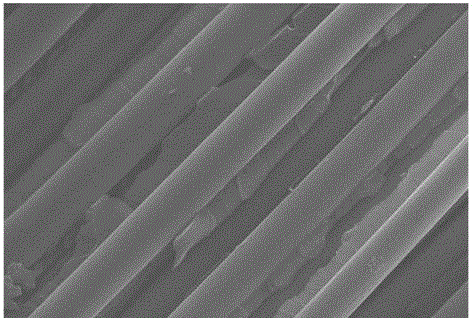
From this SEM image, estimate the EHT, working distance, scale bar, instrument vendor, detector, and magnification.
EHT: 20 kV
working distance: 6.4 mm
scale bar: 10000 nm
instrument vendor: Zeiss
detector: InLens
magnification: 5 K X